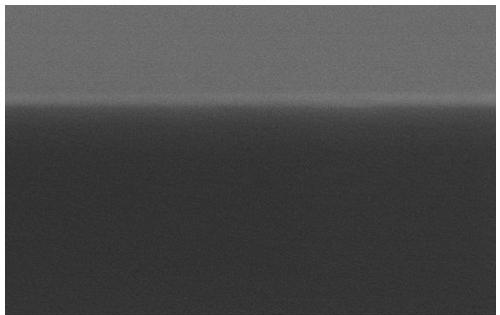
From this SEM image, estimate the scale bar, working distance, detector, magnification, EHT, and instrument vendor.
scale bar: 300 nm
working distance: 7.3 mm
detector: SE(M,LA3)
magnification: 150 K X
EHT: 3 kV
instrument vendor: Hitachi